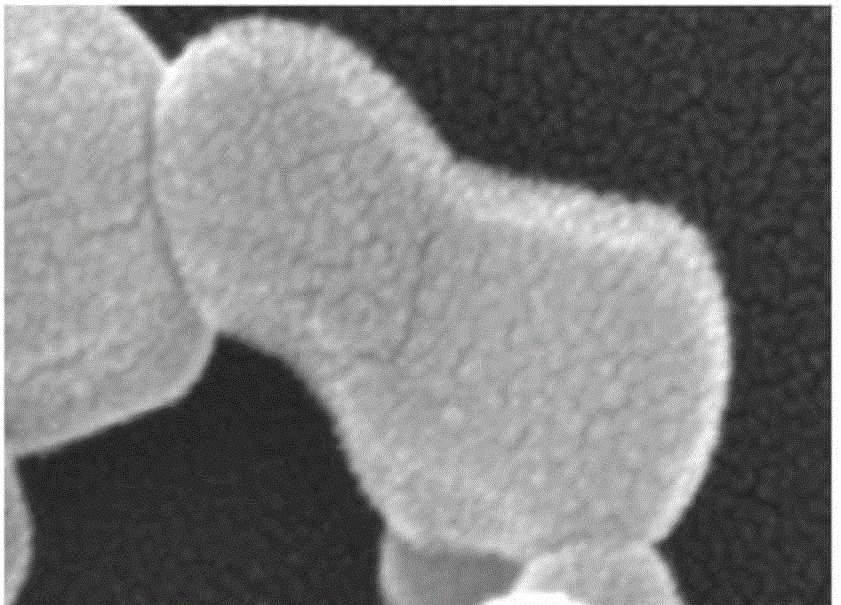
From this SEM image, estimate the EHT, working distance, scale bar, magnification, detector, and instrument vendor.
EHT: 15 kV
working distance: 4.9 mm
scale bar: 200 nm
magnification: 800 K X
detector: TLD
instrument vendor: FEI